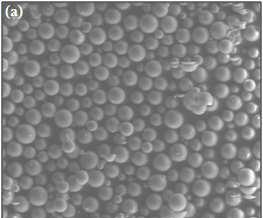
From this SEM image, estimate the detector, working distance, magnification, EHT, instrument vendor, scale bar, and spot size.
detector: LFD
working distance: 10.6 mm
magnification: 0.1 K X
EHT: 15 kV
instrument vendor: FEI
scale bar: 500000 nm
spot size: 5